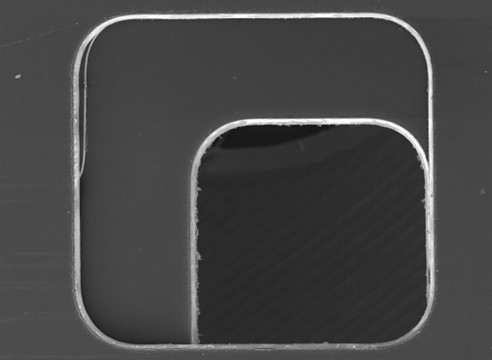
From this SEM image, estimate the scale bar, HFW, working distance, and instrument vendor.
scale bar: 500000 nm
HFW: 2750 µm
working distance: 12.91 mm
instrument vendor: Tescan